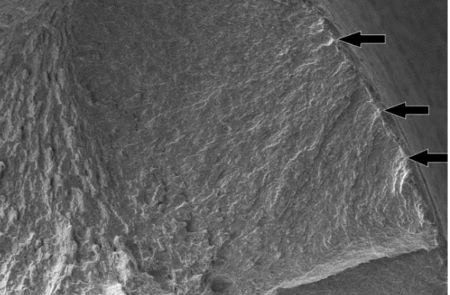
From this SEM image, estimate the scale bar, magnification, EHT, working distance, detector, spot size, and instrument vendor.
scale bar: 100000 nm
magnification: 0.2 K X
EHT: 20 kV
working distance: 11.5 mm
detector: SE1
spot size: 378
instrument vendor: Zeiss